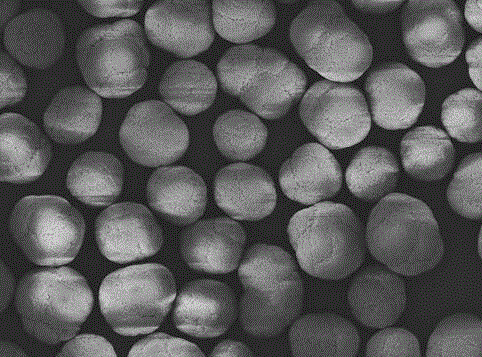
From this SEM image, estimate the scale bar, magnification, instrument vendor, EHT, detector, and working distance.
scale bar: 10000 nm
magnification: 1 K X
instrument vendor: JEOL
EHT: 15 kV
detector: ADD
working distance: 8.1 mm